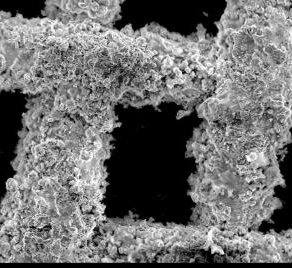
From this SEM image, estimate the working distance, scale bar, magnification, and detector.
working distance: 13.6 mm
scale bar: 100000 nm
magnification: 0.15 K X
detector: SE